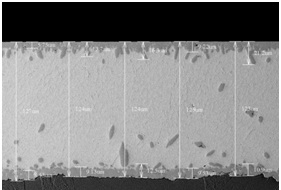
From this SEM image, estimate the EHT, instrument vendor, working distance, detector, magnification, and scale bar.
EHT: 15 kV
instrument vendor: Hitachi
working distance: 6.8 mm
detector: BSE3D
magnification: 0.5 K X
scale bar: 100000 nm